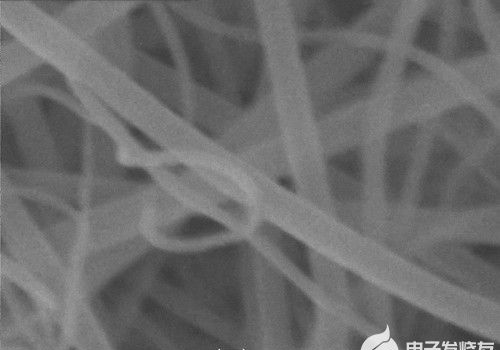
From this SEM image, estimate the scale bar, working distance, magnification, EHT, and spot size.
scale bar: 100 nm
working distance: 5.9 mm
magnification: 100 K X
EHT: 20 kV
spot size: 5